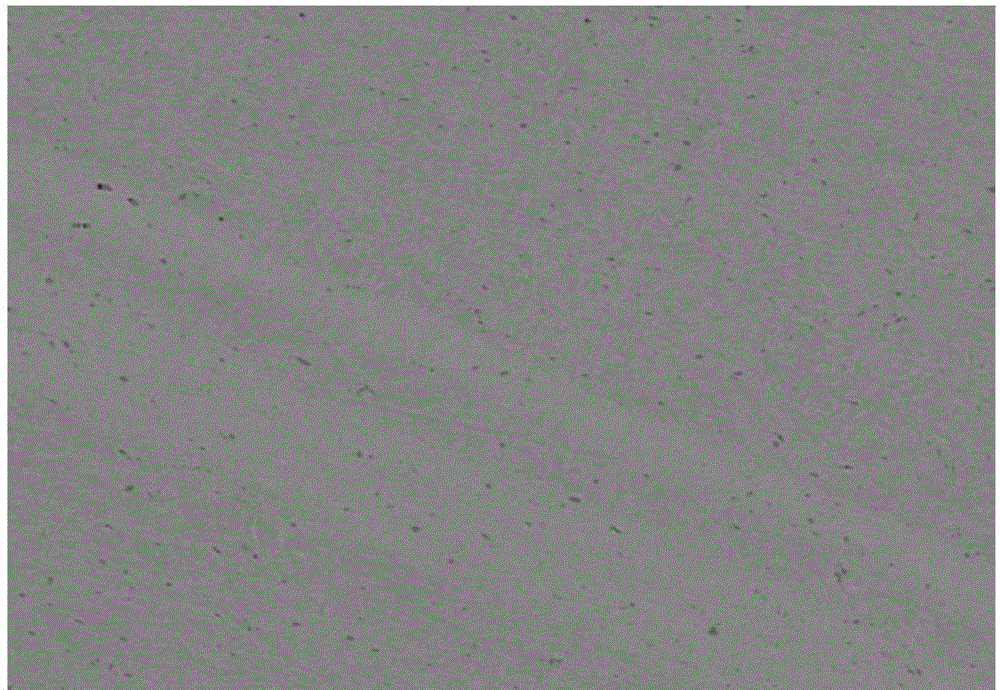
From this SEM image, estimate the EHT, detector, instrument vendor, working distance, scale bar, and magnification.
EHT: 15 kV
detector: AsB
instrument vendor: Zeiss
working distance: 6.1 mm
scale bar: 10000 nm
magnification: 1 K X